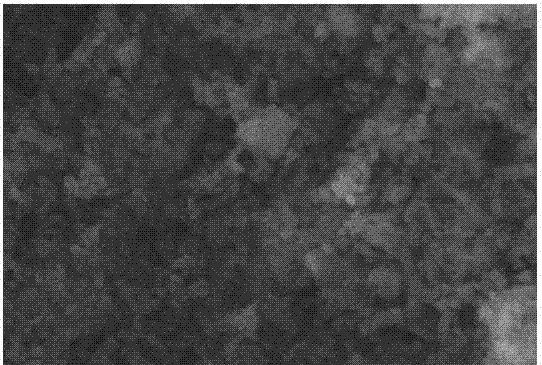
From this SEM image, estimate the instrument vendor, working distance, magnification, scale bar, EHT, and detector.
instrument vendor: JEOL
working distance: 10 mm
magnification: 4.5 K X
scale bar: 5000 nm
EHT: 20 kV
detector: SEI